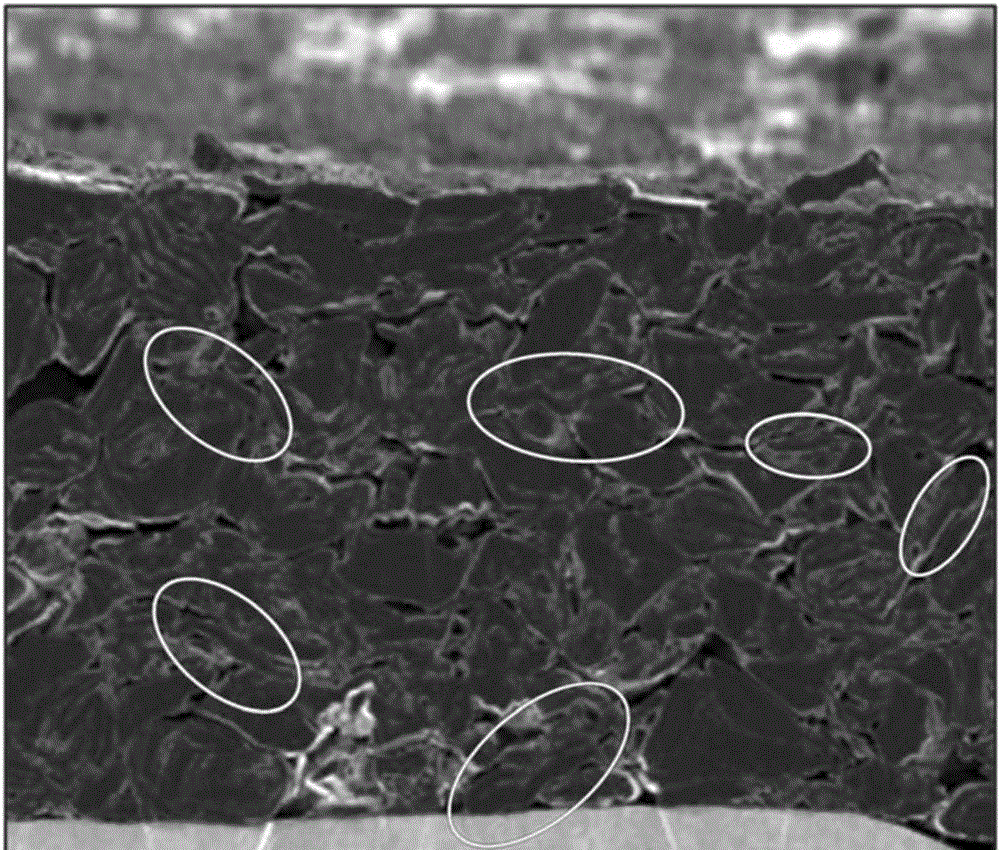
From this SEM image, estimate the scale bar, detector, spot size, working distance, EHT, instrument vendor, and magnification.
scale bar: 30000 nm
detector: ETD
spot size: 4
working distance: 6.4 mm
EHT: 3 kV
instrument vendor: FEI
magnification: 2.5 K X